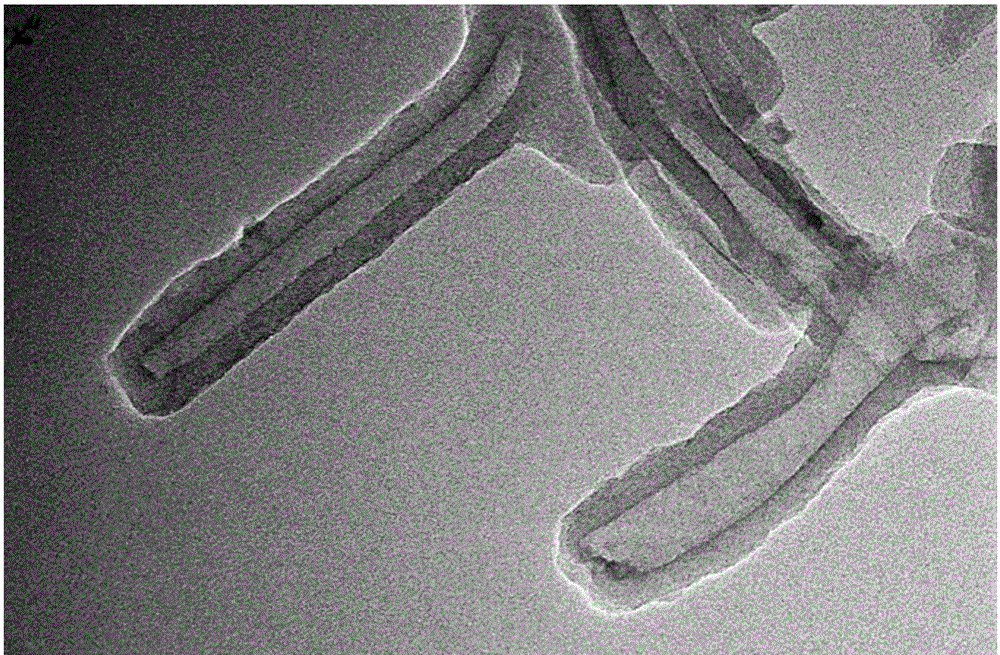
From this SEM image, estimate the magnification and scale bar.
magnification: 40 K X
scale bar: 100 nm